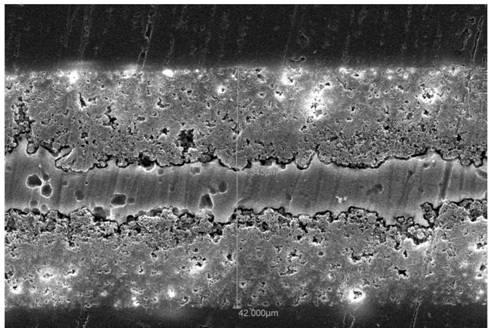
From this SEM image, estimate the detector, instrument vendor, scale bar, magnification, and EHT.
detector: SEI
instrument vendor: JEOL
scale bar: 20000 nm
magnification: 0.6 K X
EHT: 30 kV